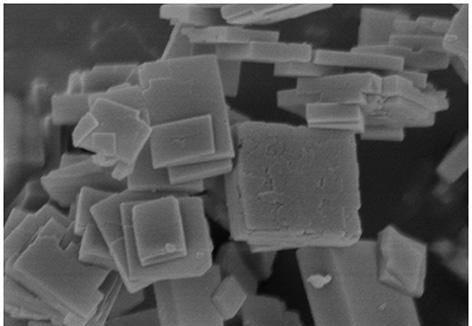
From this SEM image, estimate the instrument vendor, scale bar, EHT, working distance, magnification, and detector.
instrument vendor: Hitachi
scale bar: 1000 nm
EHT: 3 kV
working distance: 6.1 mm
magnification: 50 K X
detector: SE(M)